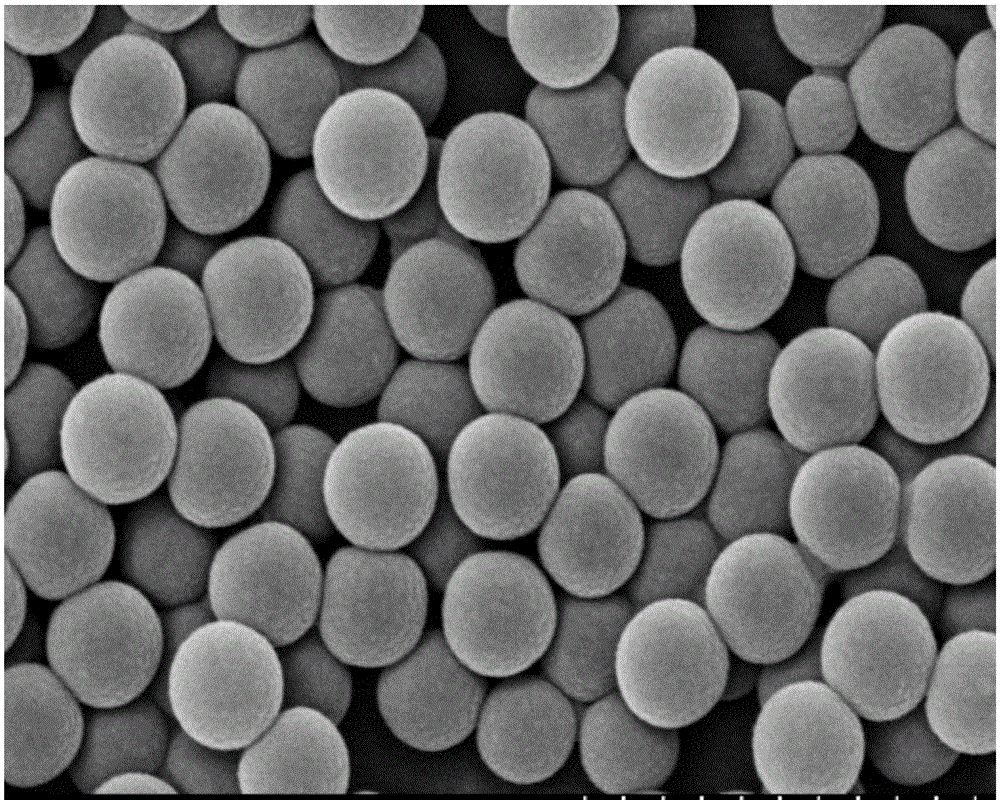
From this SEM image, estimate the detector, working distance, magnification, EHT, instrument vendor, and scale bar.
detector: SE(M)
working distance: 7.4 mm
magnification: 50 K X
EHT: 15 kV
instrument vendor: Hitachi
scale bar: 1000 nm